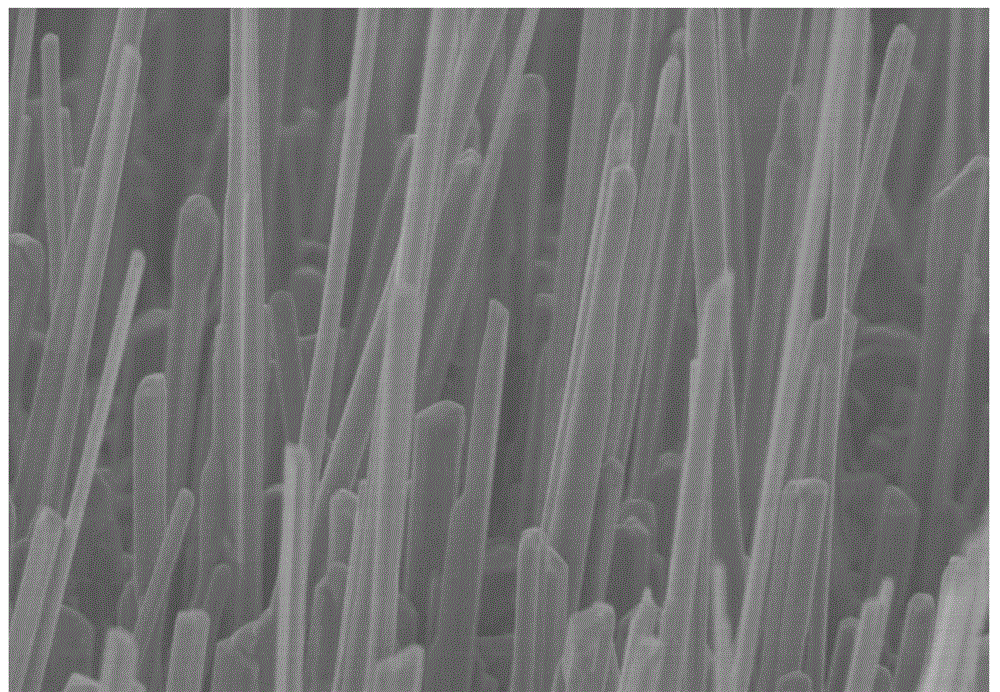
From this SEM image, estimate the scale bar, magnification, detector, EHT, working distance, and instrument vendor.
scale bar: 5000 nm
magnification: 10 K X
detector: SE(M)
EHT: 5 kV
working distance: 10.1 mm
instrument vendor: Hitachi